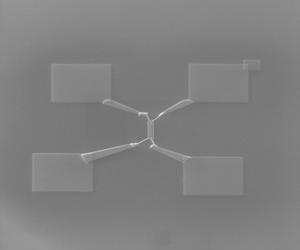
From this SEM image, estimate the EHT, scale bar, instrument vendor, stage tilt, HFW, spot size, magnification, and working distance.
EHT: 30 kV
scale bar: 20000 nm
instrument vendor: FEI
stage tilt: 52°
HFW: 122 µm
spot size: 2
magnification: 2.5 K X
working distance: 5.084 mm